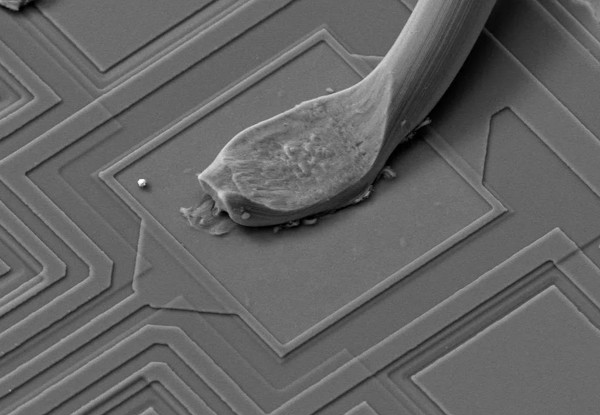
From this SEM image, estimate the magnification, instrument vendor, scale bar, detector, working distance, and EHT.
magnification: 1 K X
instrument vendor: Zeiss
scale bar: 10000 nm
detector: SE1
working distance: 14.5 mm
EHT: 20 kV